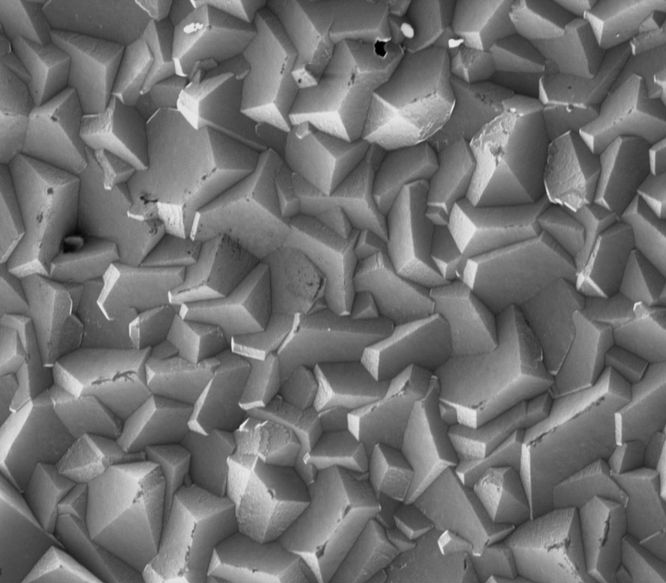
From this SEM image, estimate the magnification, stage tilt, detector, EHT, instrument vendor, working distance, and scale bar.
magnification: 6.01 K X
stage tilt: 0°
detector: ETD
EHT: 30 kV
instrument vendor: FEI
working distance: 14.5 mm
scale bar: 10000 nm